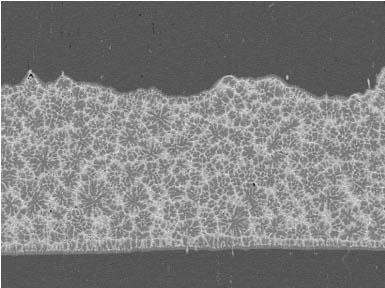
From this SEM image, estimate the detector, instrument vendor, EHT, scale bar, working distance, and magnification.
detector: COMPO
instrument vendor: JEOL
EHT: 15 kV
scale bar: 100000 nm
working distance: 14.9 mm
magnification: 0.2 K X